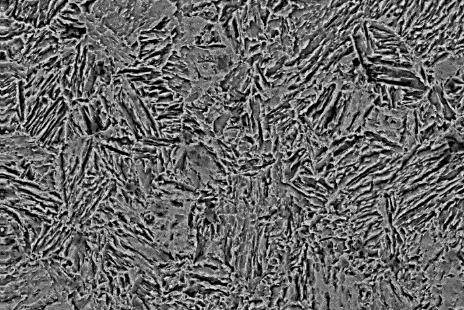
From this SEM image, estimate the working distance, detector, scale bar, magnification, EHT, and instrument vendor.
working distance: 8.5 mm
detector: CZ BSD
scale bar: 10000 nm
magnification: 3 K X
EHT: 20 kV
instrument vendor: Zeiss